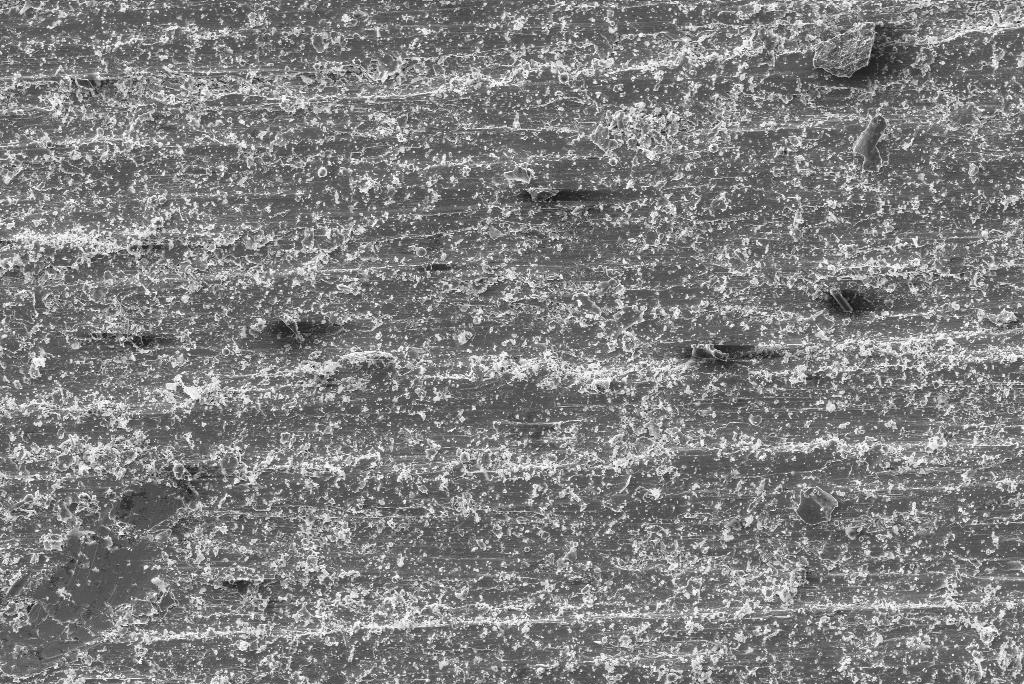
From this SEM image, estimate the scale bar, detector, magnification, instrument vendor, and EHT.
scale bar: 100000 nm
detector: SE1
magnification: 0.1 K X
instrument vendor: Zeiss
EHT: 20 kV